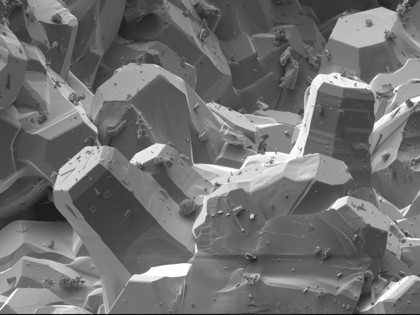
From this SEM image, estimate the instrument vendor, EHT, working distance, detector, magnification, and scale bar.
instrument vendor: JEOL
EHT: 5 kV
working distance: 24.2 mm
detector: LEI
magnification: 0.2 K X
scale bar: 100000 nm